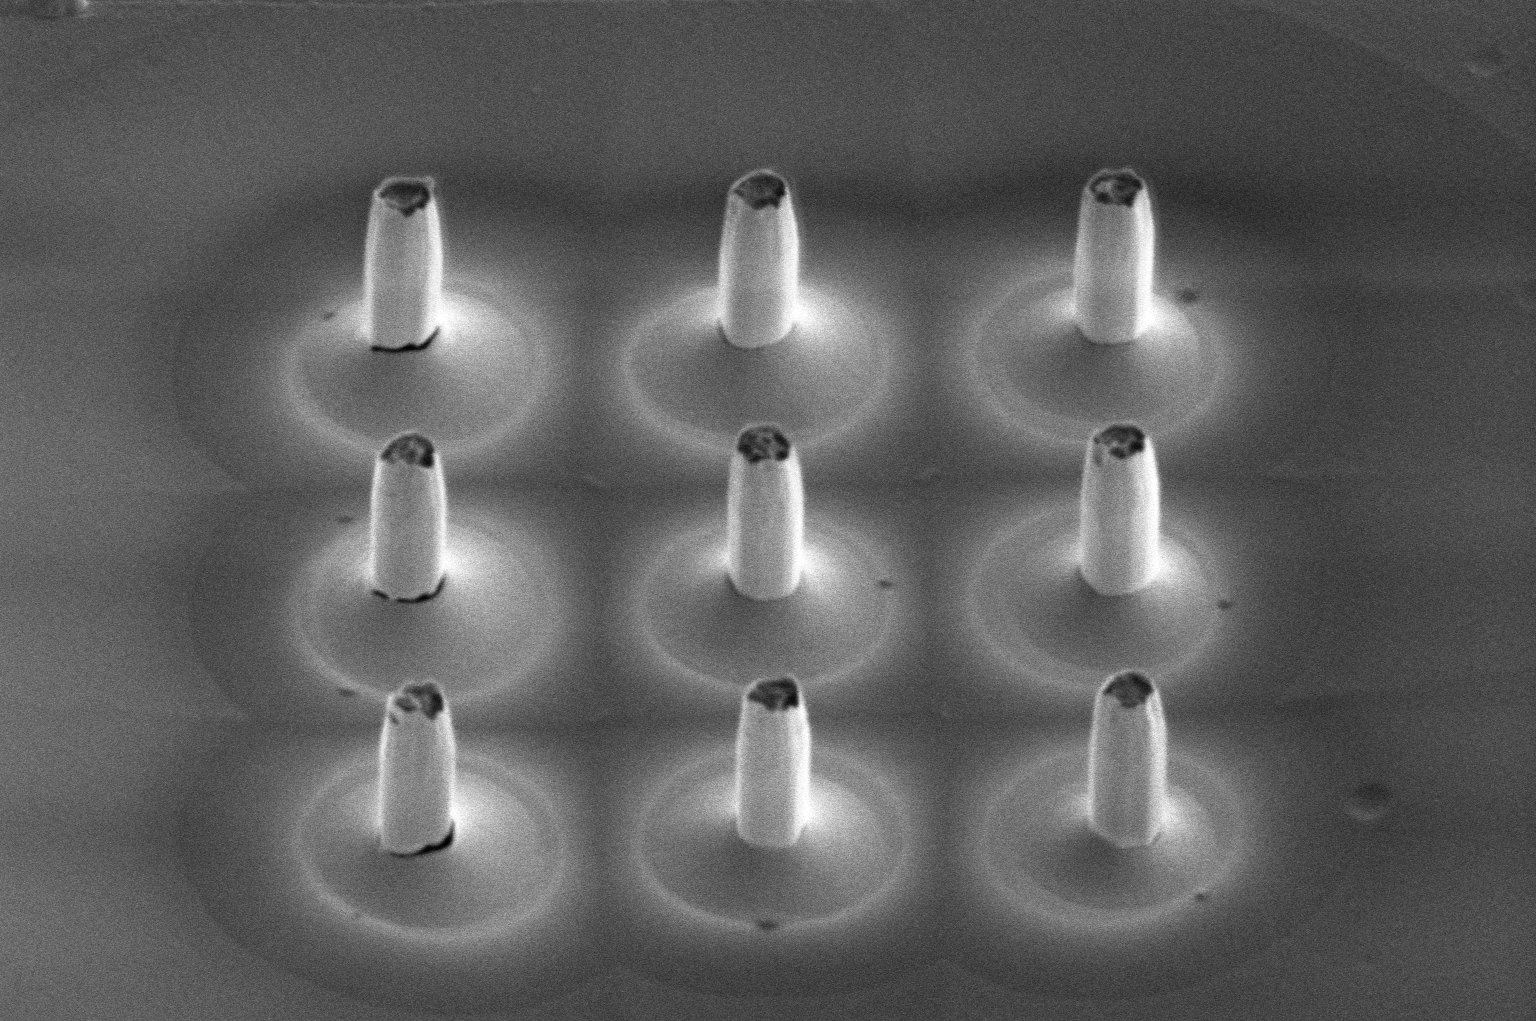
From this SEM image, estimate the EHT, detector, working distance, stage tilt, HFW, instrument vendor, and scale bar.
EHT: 2 kV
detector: ETD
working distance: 10.2 mm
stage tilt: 45°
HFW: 25.9 µm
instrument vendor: Thermo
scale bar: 5000 nm